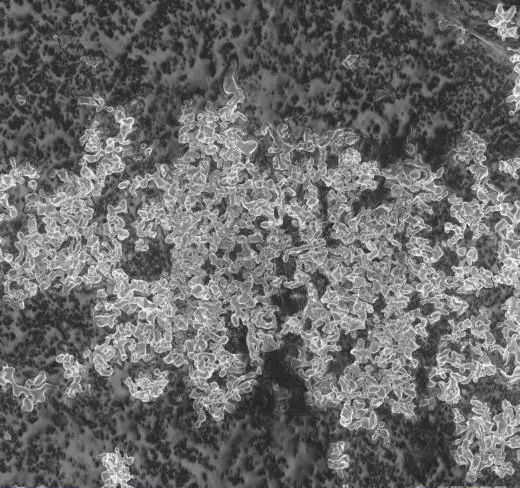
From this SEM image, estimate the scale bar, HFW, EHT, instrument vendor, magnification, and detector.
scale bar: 10000 nm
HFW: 145 µm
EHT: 2 kV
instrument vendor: other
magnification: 2 K X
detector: SE